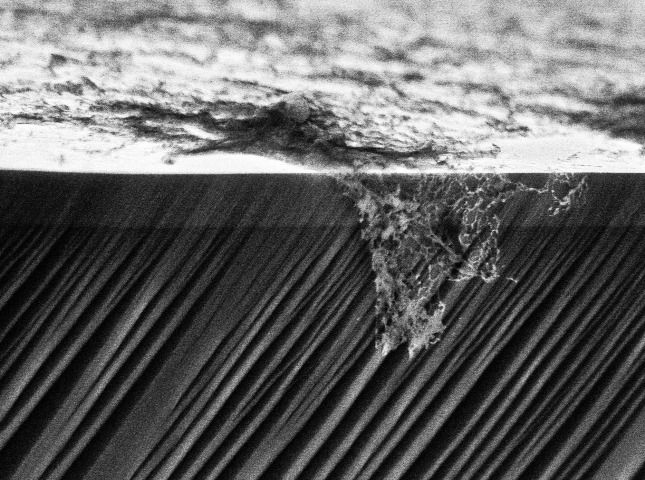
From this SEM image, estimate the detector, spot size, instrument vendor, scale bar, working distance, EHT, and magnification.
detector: TLD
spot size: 2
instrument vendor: FEI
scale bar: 1000 nm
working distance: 4.2 mm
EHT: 5 kV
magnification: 19.302 K X